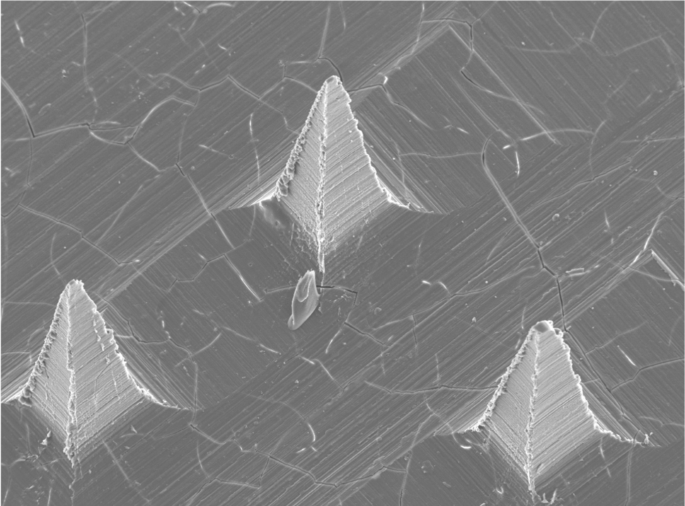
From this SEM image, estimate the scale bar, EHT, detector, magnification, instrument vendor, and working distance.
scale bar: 100000 nm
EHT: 20 kV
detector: SEI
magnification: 0.085 K X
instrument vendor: JEOL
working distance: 14.8 mm